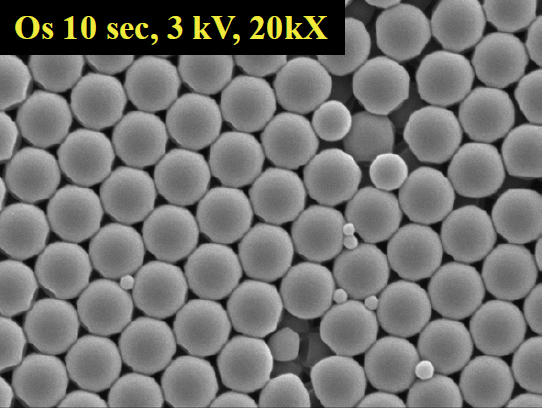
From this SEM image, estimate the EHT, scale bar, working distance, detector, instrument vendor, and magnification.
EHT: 3 kV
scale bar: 1000 nm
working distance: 10 mm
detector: SEI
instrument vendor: JEOL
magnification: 20 K X